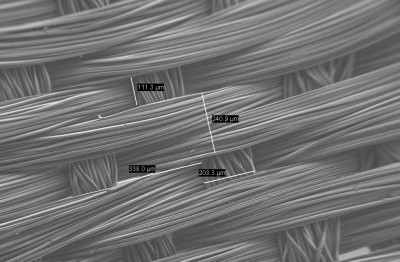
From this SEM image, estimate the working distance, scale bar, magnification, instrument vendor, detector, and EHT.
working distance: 6.2 mm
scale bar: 100000 nm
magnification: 0.178 K X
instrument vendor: Zeiss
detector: SE2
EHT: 1 kV